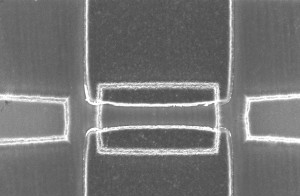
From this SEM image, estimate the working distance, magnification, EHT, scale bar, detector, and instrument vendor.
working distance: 8.1 mm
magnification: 9.97 K X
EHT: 5 kV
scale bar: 2000 nm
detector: InLens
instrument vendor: Zeiss